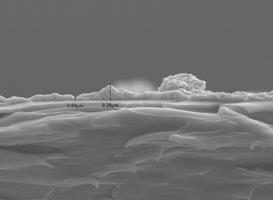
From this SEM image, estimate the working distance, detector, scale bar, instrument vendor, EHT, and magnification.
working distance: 10.3 mm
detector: SEI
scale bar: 10000 nm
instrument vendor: JEOL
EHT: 15 kV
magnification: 2 K X